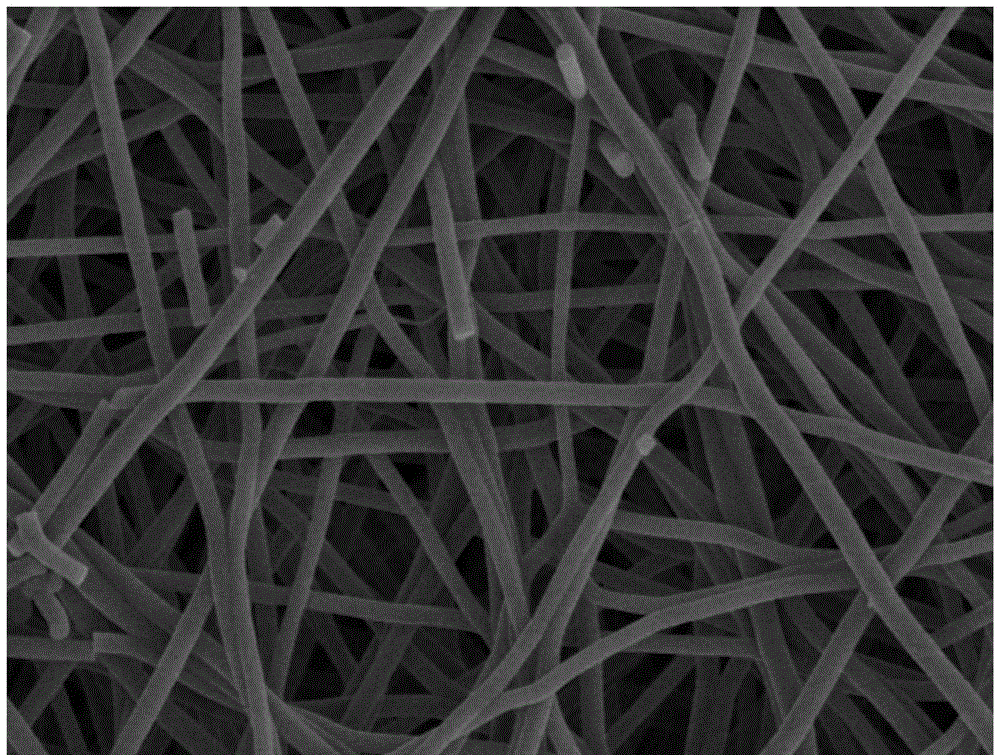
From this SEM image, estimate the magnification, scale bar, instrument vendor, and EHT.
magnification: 10 K X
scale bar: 5000 nm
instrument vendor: Hitachi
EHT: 5 kV